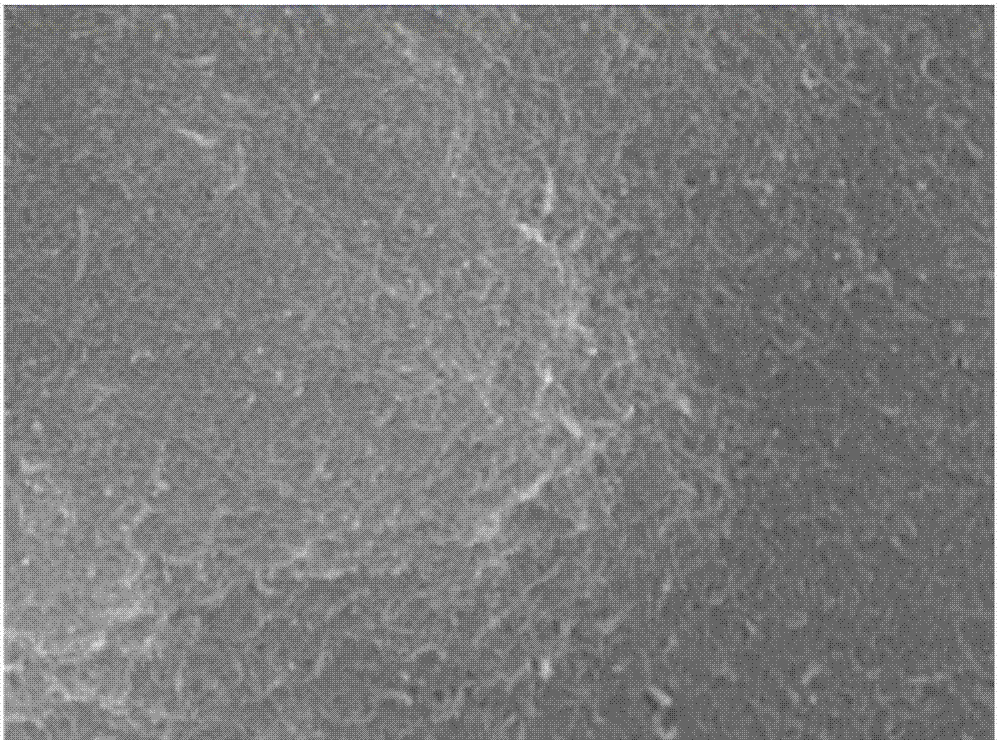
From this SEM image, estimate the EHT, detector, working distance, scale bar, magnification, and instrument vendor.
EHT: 15 kV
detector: SEI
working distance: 10.7 mm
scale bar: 100 nm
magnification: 30 K X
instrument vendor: JEOL